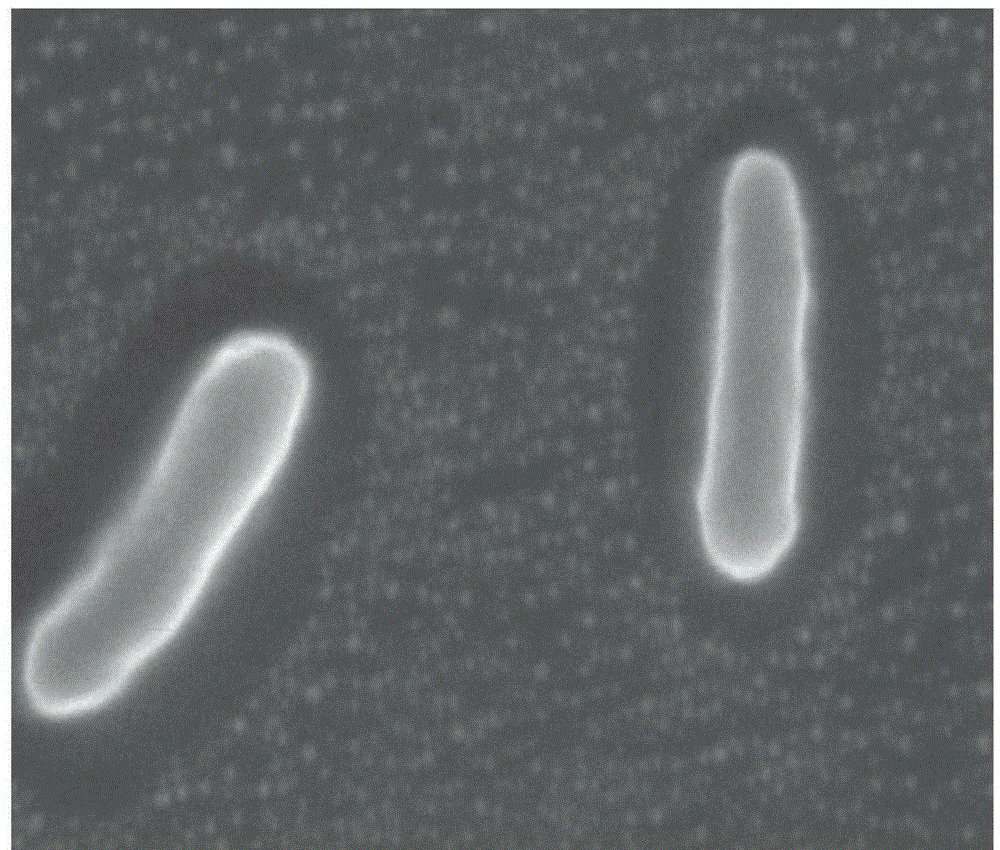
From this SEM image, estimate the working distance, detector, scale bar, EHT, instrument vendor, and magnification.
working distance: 9 mm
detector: SE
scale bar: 1000 nm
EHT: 15 kV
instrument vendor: Hitachi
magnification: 27 K X